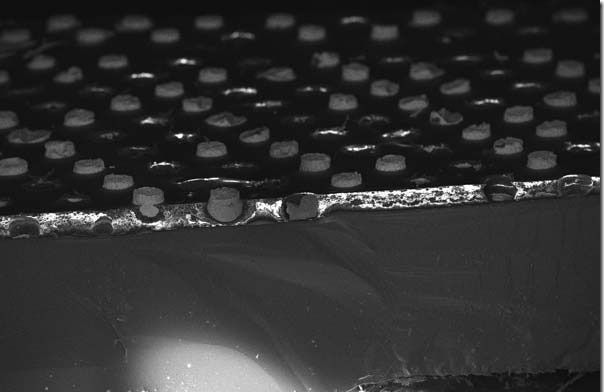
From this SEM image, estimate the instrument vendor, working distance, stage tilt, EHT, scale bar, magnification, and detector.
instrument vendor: Zeiss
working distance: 4 mm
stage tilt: -15°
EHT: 15 kV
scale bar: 200000 nm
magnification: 0.113 K X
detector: InLens